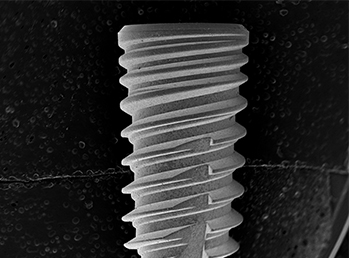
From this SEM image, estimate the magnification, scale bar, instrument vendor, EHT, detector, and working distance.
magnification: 0.017 K X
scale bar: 3000000 nm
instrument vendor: other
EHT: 15 kV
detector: SE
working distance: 42.7 mm